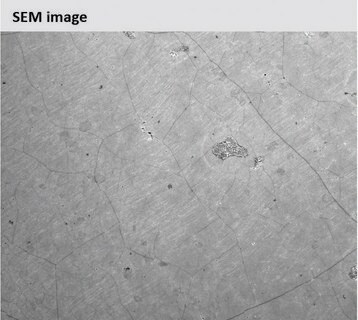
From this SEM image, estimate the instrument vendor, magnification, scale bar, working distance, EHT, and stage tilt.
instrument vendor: FEI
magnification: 8 K X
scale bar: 10000 nm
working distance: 3.1 mm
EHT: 1 kV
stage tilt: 0°